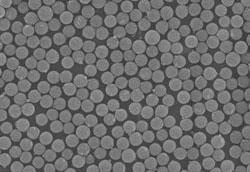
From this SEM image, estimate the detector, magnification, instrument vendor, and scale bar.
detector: SE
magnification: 10 K X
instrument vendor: Hitachi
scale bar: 5000 nm